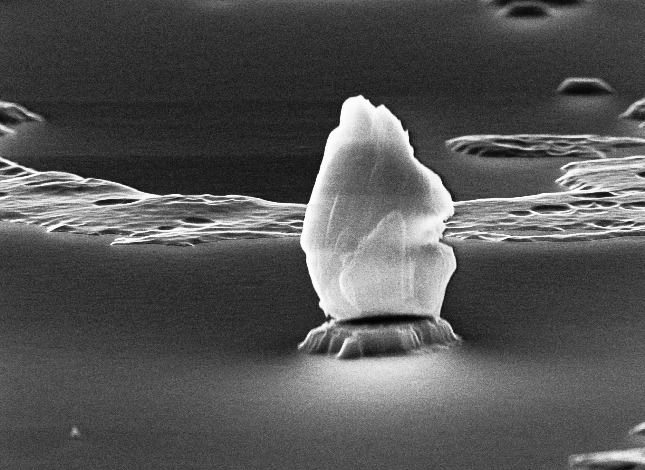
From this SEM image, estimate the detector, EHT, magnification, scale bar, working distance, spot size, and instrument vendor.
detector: SE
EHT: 30 kV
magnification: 16.119 K X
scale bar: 1000 nm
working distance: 15 mm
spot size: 4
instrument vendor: FEI